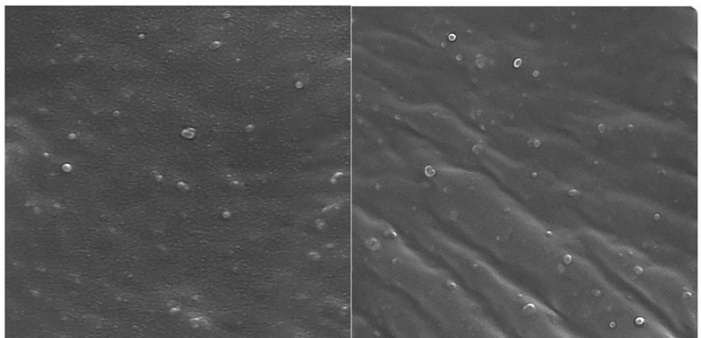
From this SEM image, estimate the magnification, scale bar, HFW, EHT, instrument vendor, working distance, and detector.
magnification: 50 K X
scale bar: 500 nm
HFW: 2.89 µm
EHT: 15 kV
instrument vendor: Tescan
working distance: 6.76 mm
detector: InBeam